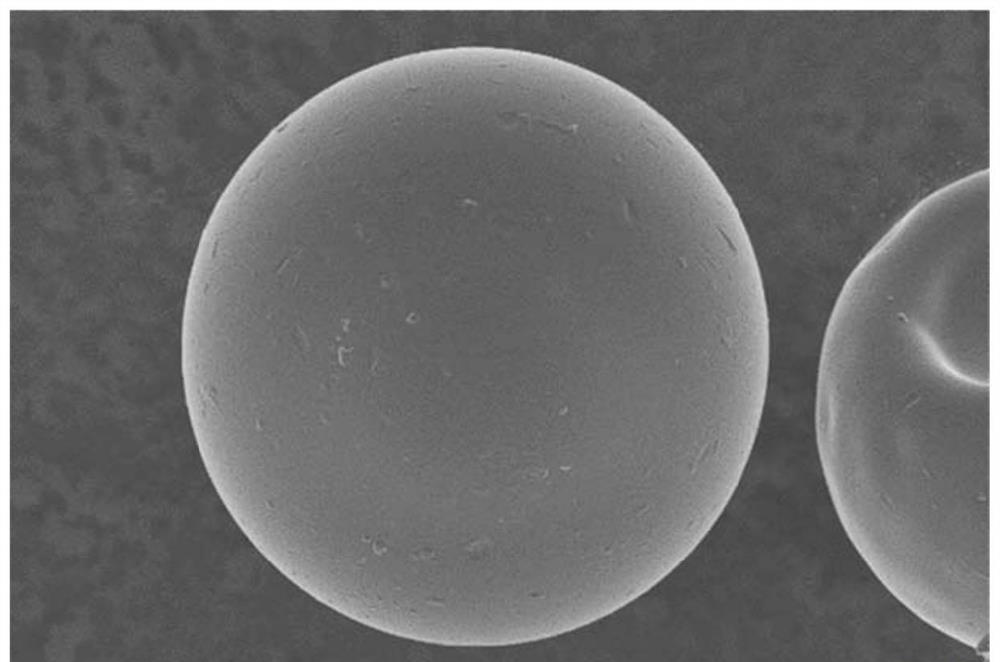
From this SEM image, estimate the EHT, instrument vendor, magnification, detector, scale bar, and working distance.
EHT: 3 kV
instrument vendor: Zeiss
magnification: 1.37 K X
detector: InLens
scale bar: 10000 nm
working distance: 6.2 mm